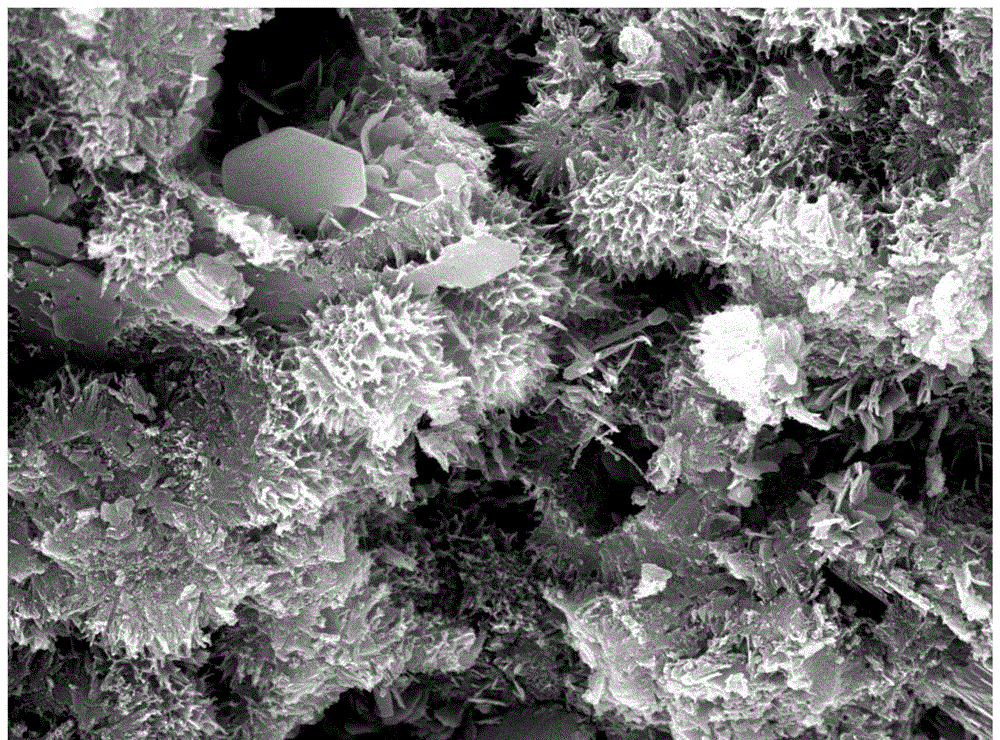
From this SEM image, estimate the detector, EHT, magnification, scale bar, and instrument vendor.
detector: SED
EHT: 20 kV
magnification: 5 K X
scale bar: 5000 nm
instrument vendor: JEOL